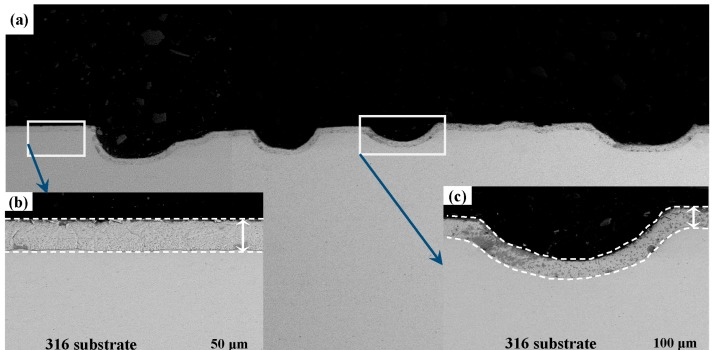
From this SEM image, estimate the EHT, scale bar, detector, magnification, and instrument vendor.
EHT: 20 kV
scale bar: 1000000 nm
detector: BSE Detector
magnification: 0.1 K X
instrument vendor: Tescan